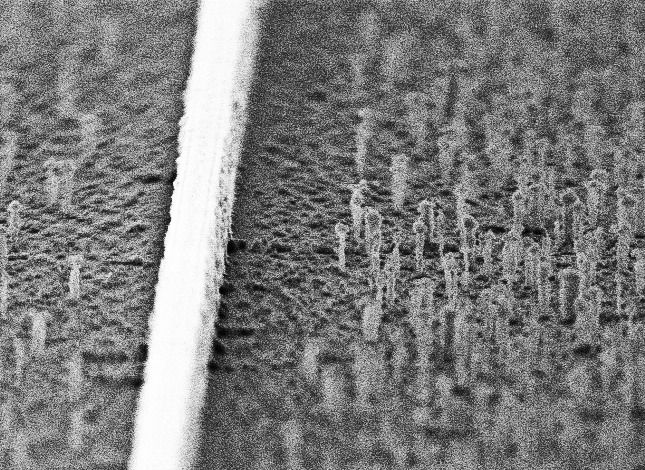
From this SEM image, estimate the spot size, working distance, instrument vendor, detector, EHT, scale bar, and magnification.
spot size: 3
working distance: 12 mm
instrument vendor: FEI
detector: SE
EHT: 10 kV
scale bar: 1000 nm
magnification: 29.064 K X